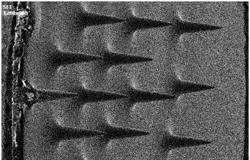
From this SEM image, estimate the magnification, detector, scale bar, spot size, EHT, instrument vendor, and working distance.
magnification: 0.05 K X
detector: SEI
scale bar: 100000 nm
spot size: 13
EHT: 20 kV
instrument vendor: other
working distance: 49.9 mm